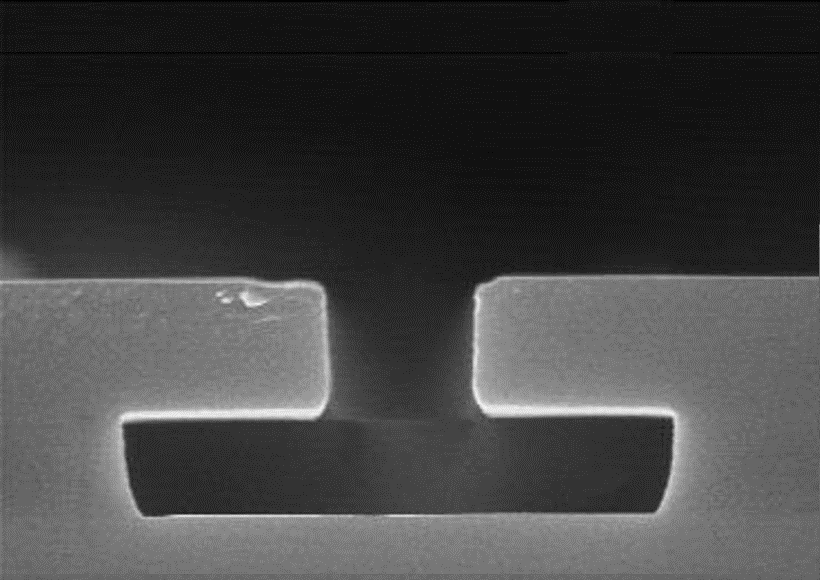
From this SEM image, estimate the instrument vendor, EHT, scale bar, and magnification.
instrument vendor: JEOL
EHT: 15 kV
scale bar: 1200 nm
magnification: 25 K X